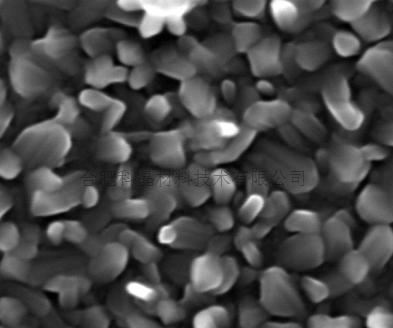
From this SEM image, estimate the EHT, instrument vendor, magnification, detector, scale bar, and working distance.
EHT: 20 kV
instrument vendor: FEI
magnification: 80 K X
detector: TLD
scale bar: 500 nm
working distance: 6 mm